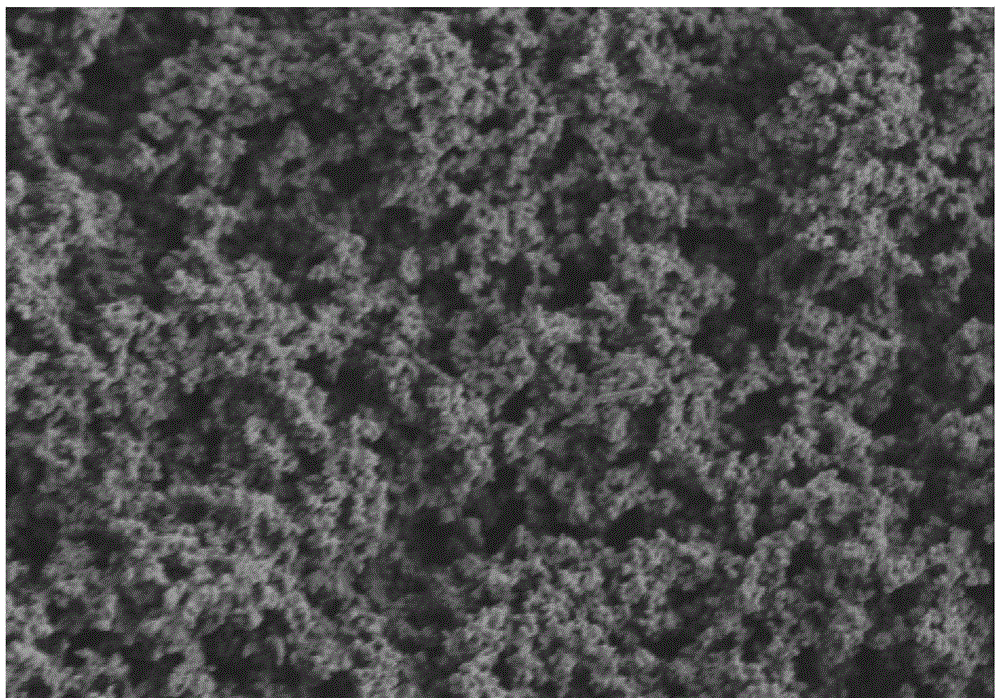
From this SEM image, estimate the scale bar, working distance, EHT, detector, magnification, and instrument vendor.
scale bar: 10000 nm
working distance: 8.9 mm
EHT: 2 kV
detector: SE(L)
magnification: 5 K X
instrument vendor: Hitachi